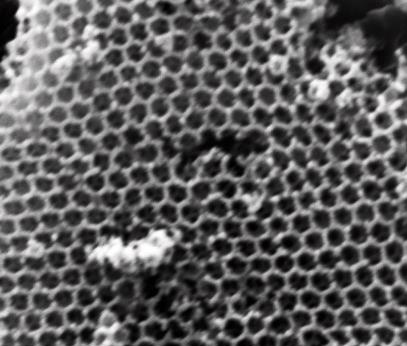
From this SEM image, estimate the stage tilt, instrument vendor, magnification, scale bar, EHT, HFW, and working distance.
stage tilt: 1°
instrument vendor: FEI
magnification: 50 K X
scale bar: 1000 nm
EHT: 25 kV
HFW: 5.41 µm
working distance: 10.6 mm